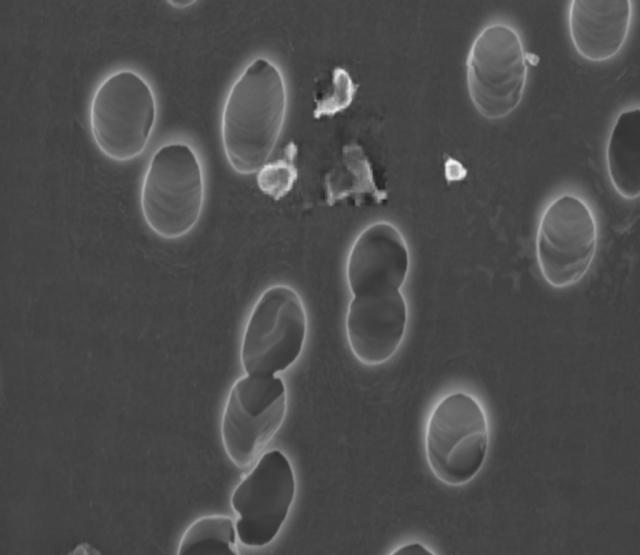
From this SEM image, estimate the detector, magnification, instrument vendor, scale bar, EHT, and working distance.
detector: InLens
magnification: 40 K X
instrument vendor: Zeiss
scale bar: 1000 nm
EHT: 3 kV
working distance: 3.1 mm